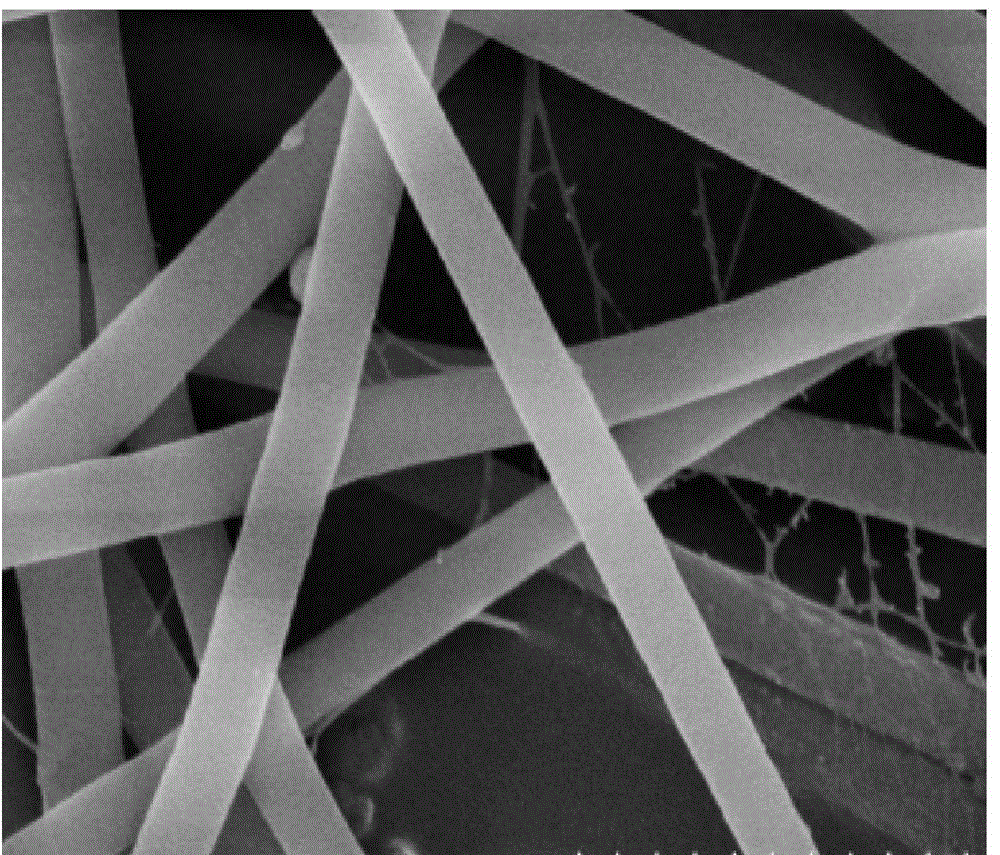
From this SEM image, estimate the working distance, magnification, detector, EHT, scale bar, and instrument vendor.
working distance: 7.7 mm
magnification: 50 K X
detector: SE(M)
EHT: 5 kV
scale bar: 1000 nm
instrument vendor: Hitachi